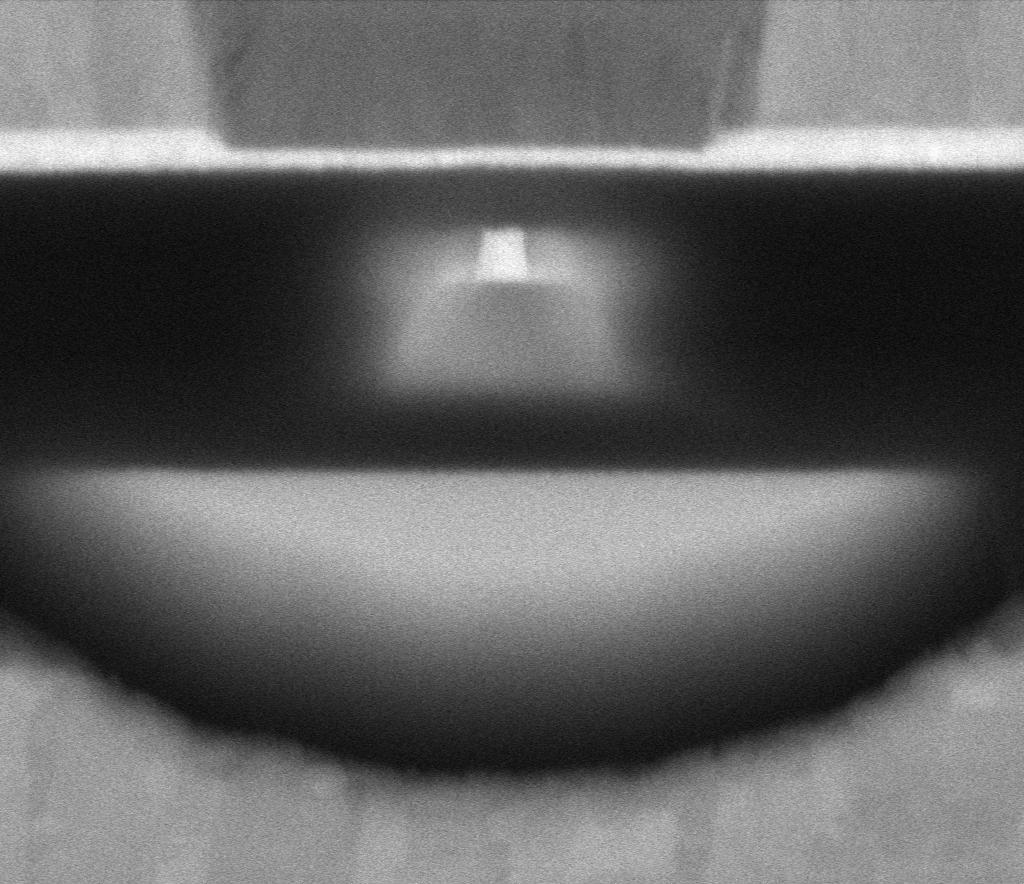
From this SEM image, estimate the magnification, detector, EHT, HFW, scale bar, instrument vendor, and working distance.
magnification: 568.609 K X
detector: MD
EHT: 5 kV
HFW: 0.635 µm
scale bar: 100 nm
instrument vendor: Thermo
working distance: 2 mm